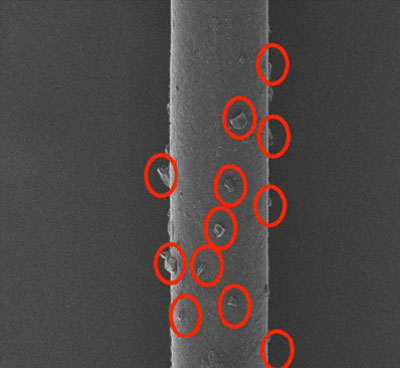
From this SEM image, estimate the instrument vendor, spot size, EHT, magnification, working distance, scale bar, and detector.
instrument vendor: JEOL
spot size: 40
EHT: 5 kV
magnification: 0.3 K X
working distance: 9 mm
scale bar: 50000 nm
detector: SEI